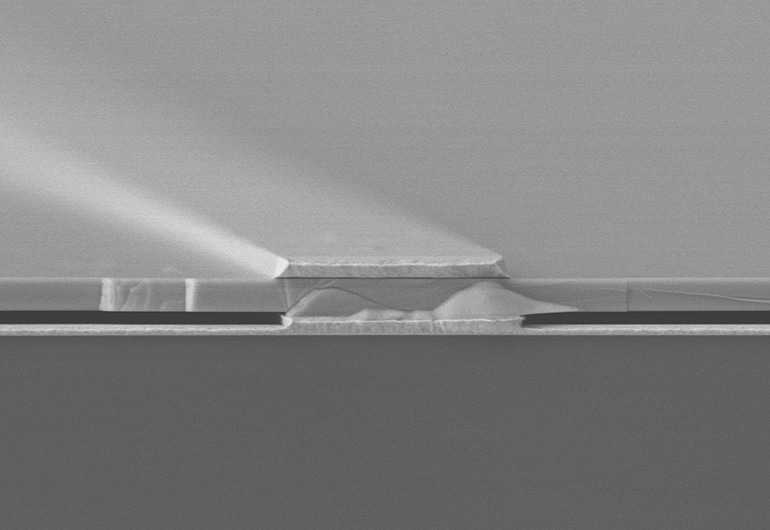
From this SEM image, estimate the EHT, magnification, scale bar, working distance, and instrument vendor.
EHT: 10 kV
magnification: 10 K X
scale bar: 10000 nm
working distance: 12.5 mm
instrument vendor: Hitachi